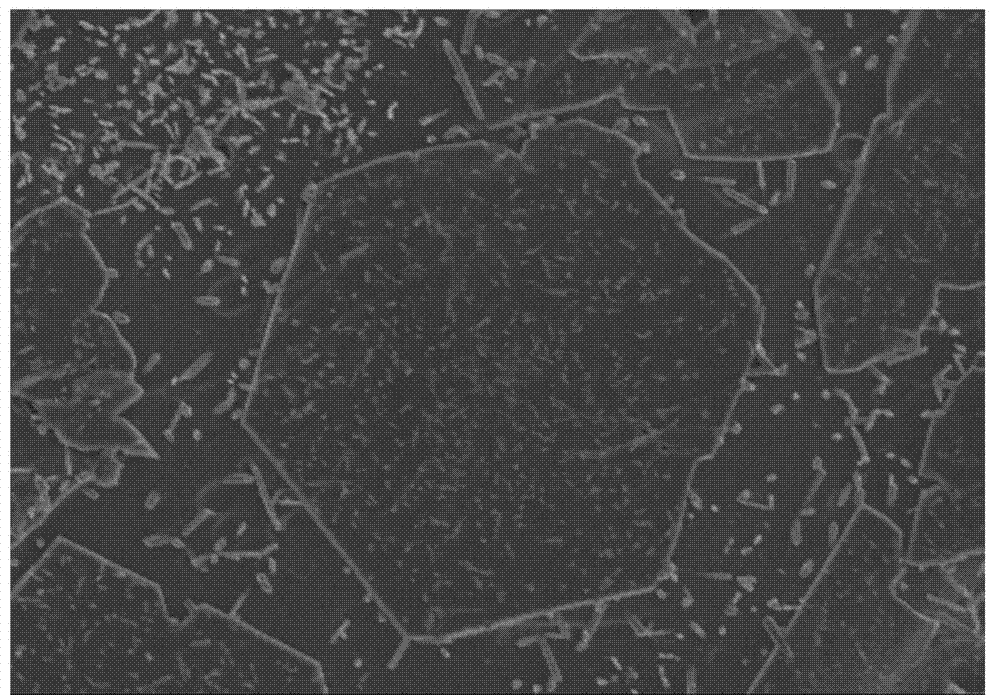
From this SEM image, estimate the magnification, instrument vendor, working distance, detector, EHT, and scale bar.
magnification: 3 K X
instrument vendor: Hitachi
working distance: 9.3 mm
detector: SE(M)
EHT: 5 kV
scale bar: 10000 nm